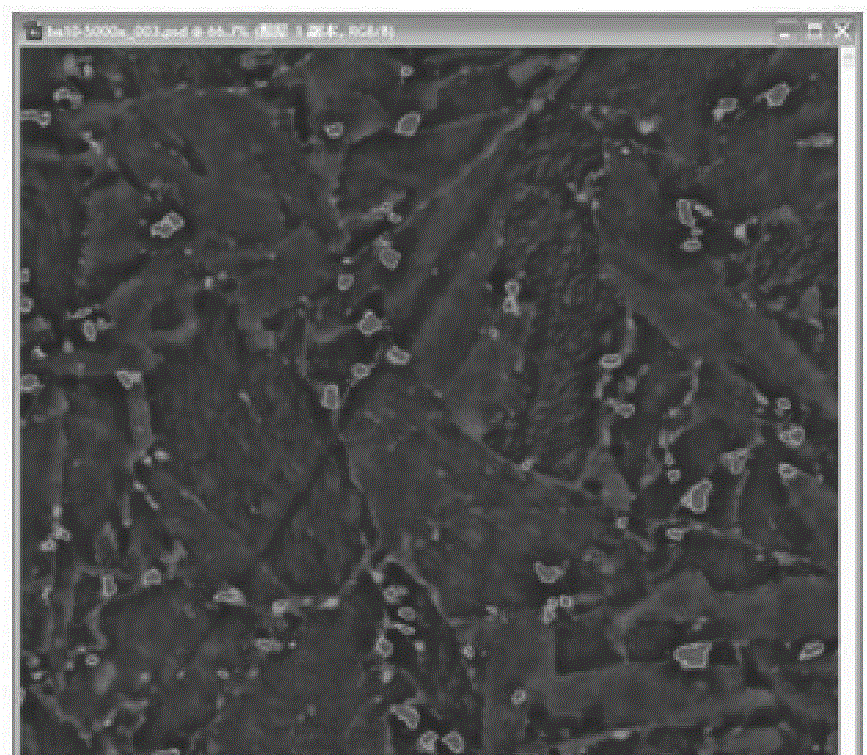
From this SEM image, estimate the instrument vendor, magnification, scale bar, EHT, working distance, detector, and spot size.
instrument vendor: FEI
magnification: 5 K X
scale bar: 10000 nm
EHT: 20 kV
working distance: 8 mm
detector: SSD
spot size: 4.5